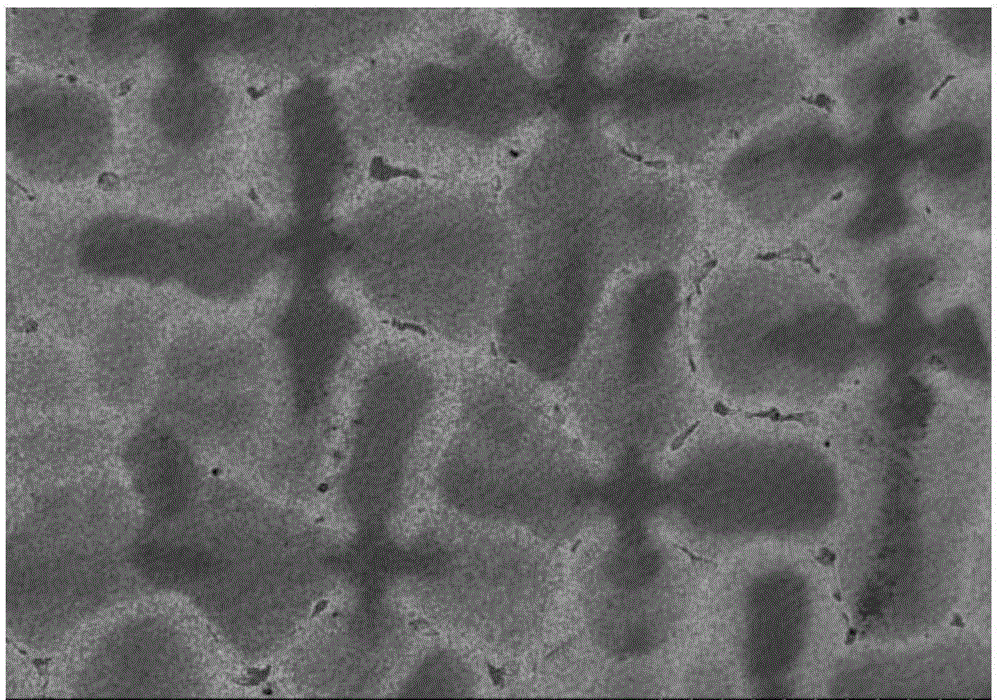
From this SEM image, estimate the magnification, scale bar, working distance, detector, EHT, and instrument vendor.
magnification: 0.2 K X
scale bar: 200000 nm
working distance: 8.7 mm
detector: SE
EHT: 20 kV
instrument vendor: Hitachi